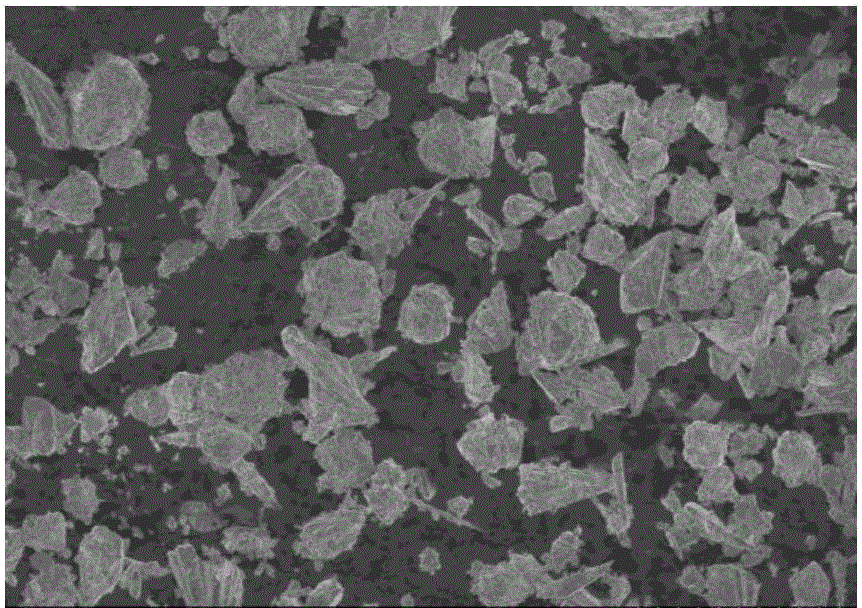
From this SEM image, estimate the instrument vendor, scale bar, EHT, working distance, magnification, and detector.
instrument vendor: Hitachi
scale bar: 50000 nm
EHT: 5 kV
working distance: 7.2 mm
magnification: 0.6 K X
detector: SE(U)